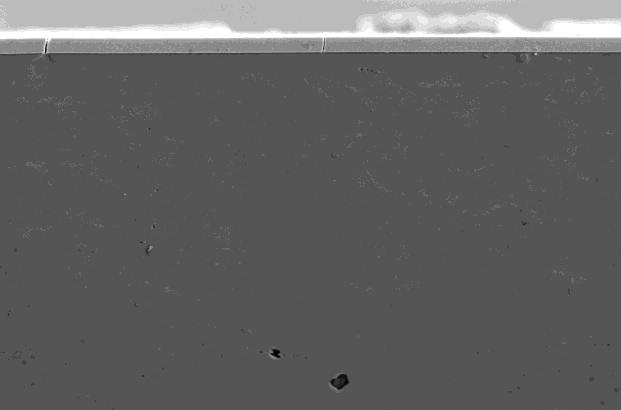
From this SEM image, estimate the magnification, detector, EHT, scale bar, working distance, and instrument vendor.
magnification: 2 K X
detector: SE1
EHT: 20 kV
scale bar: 10000 nm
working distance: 9 mm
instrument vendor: Zeiss